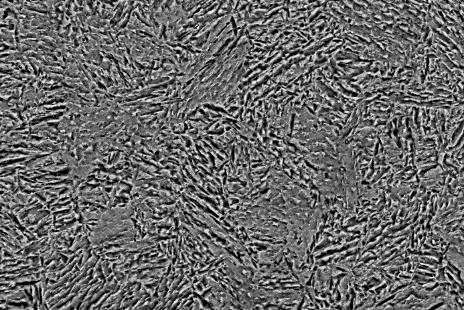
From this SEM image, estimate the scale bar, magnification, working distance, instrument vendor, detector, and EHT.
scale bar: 10000 nm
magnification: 3 K X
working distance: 8 mm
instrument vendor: Zeiss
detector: CZ BSD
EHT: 20 kV